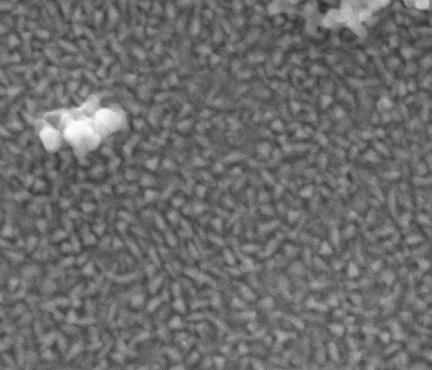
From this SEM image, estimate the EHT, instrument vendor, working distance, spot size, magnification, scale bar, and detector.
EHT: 7 kV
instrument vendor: FEI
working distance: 9.1 mm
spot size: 3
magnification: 100 K X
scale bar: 1000 nm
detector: ETD